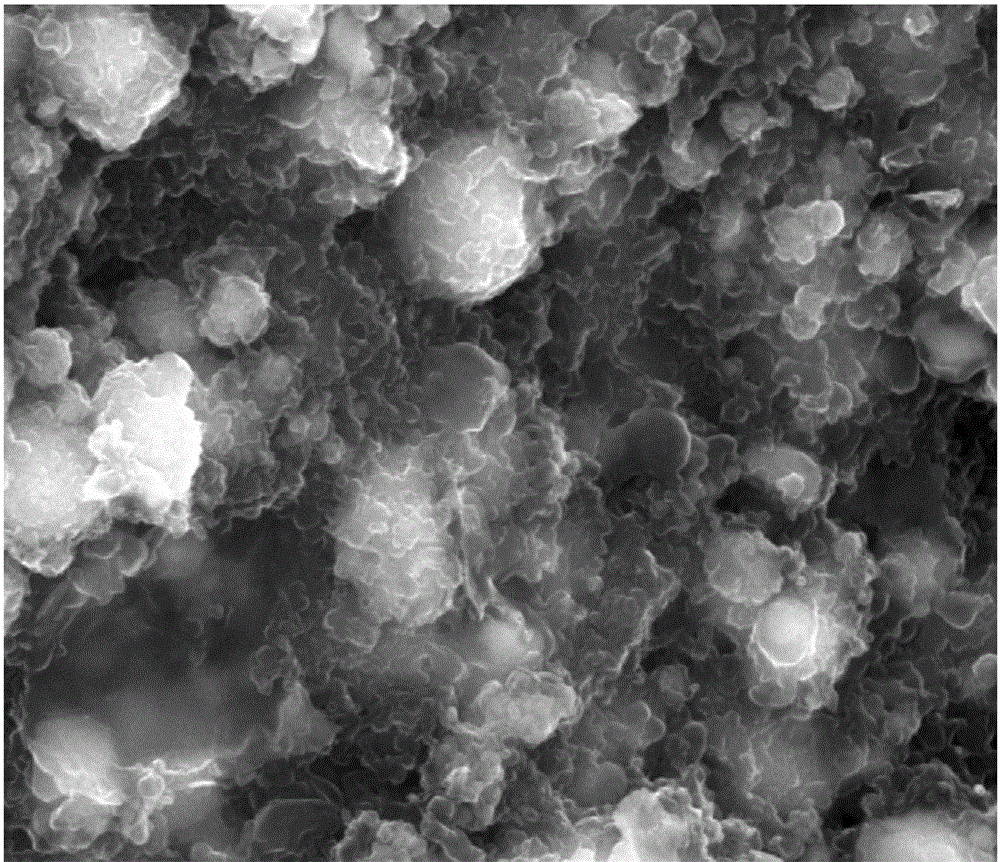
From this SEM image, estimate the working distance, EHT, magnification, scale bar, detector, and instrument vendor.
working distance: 7.3 mm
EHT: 20 kV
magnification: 50 K X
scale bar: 1000 nm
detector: SE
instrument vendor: FEI